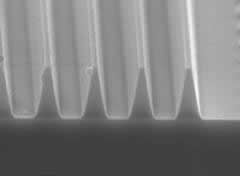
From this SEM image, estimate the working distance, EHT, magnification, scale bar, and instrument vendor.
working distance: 9.5 mm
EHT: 20 kV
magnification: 4 K X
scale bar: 2500 nm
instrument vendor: Hitachi